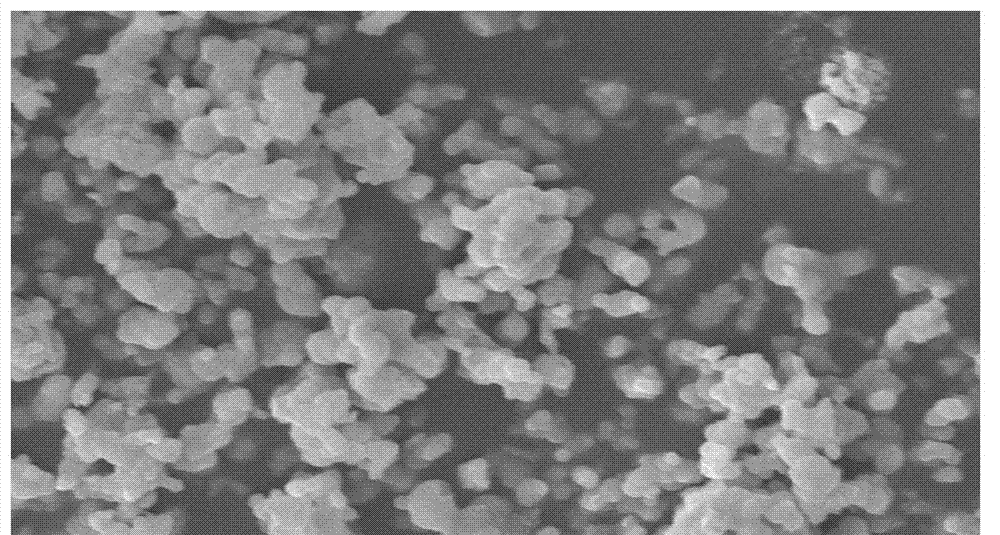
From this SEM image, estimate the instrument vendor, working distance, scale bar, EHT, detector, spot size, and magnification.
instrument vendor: FEI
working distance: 7.5 mm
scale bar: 10000 nm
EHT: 20 kV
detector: SE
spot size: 4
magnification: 4.8 K X